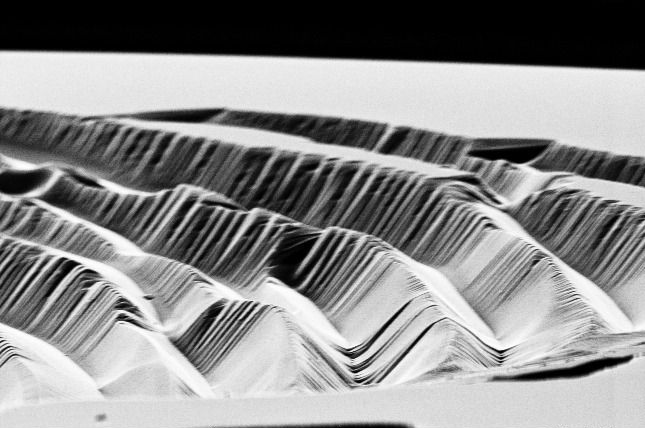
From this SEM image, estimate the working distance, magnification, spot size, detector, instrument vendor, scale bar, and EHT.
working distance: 21.9 mm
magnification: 4.788 K X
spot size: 3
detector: SE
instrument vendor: FEI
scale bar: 5000 nm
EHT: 10 kV